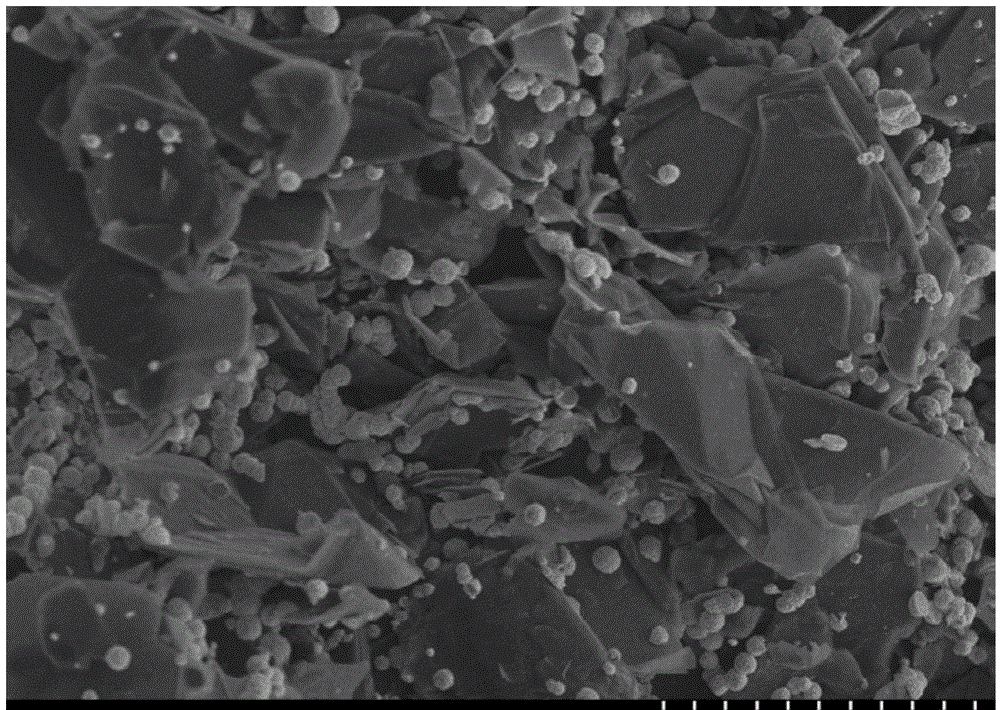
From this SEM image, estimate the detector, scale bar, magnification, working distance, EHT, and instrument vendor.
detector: SE(M)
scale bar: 10000 nm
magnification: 4 K X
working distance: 10.2 mm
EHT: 5 kV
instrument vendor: Hitachi